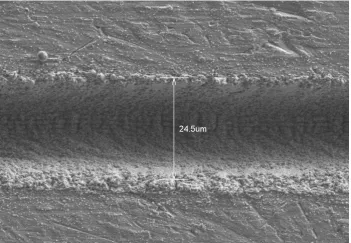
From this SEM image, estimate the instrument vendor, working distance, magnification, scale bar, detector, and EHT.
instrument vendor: Hitachi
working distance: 8 mm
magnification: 1.5 K X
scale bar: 30000 nm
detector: LM(L)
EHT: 5 kV